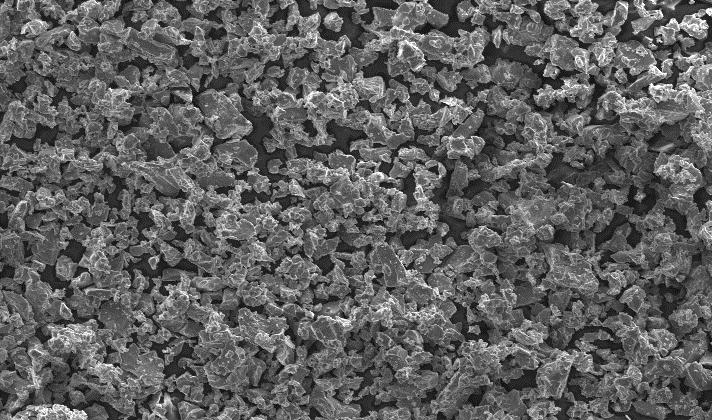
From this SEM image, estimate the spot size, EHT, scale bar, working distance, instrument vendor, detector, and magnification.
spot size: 4.3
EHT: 20 kV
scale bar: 200000 nm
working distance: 11.4 mm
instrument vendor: FEI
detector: SE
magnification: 0.1 K X